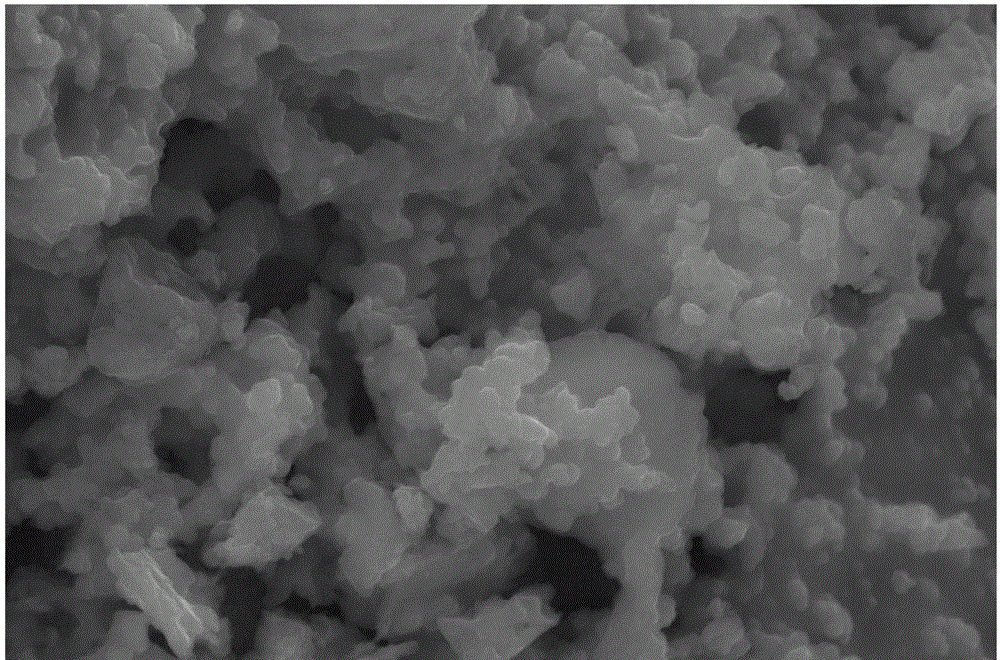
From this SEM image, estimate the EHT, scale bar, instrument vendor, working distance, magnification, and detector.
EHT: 20 kV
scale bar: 5000 nm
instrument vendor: FEI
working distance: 8.5 mm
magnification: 30 K X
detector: ETD